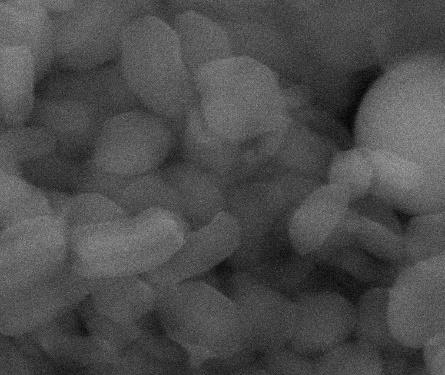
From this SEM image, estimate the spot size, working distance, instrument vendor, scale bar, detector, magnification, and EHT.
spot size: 2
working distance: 10.1 mm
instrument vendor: FEI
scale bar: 1000 nm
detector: ETD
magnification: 60 K X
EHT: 30 kV